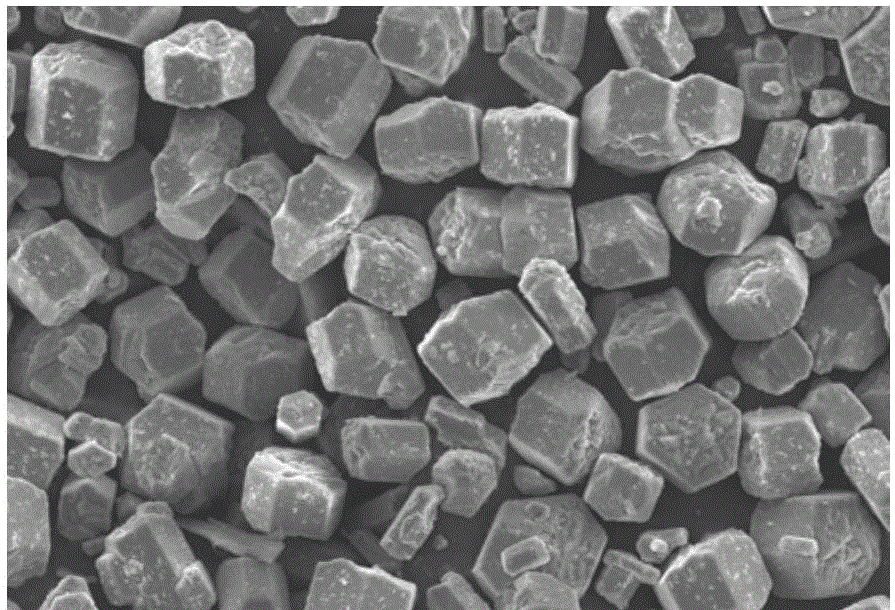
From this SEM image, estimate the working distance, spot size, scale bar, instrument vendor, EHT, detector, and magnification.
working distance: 21 mm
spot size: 30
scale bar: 50000 nm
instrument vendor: JEOL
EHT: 20 kV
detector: SEI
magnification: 0.3 K X